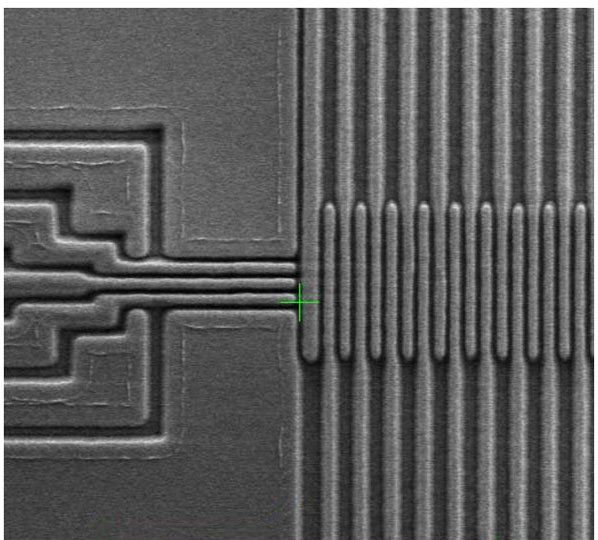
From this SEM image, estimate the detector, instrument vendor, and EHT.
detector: Int1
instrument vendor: other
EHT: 0.8 kV